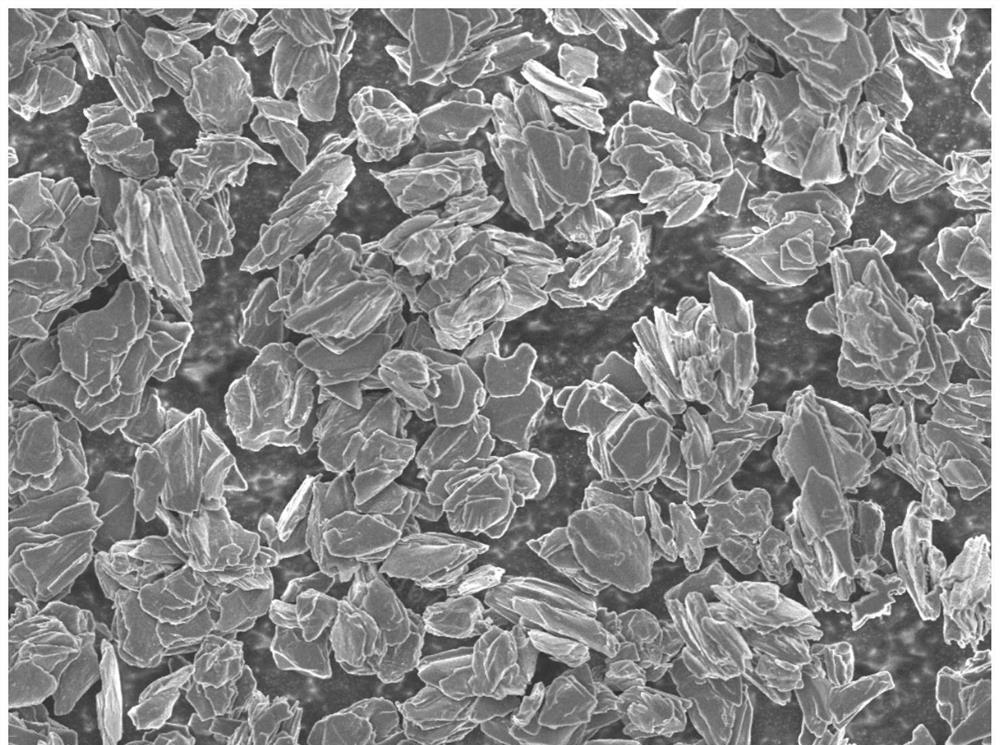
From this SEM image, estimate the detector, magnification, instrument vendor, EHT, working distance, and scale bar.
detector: SEI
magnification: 1 K X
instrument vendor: JEOL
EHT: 5 kV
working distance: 8.2 mm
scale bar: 10000 nm